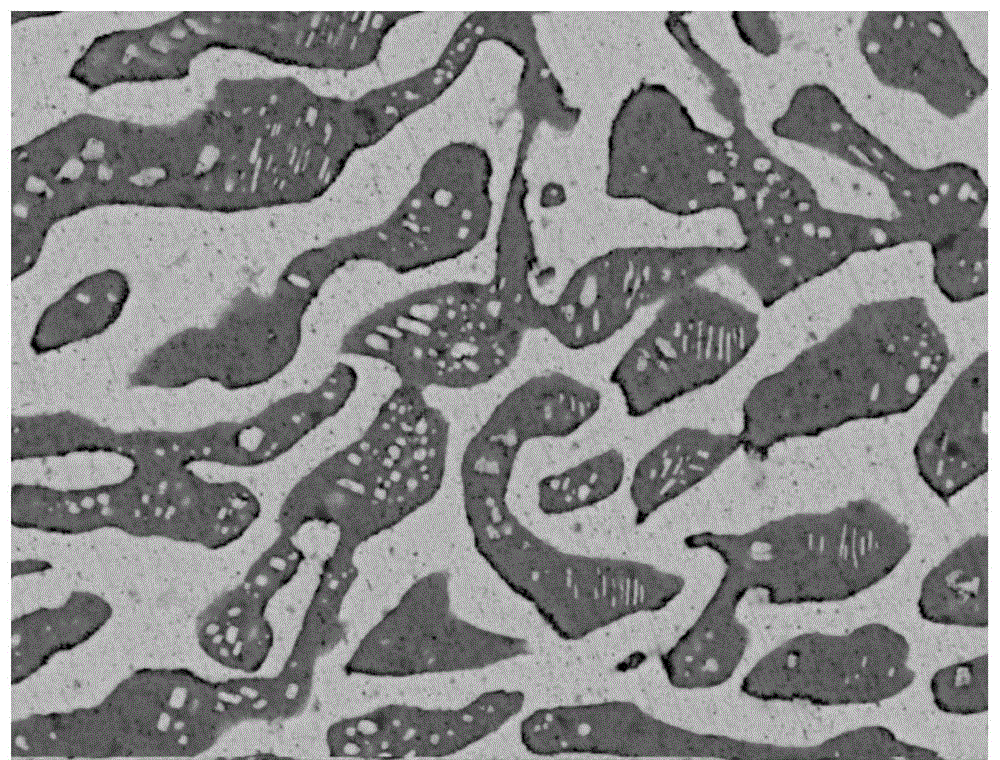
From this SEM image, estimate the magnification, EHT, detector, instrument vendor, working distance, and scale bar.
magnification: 5 K X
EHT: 20 kV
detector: vCD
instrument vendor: FEI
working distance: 12.7 mm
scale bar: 20000 nm